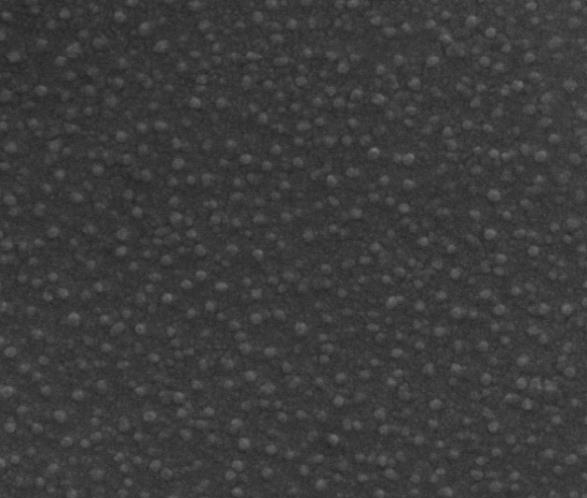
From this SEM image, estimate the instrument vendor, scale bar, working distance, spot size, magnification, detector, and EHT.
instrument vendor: FEI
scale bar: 500 nm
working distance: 10.7 mm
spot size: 3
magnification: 160 K X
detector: ETD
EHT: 20 kV